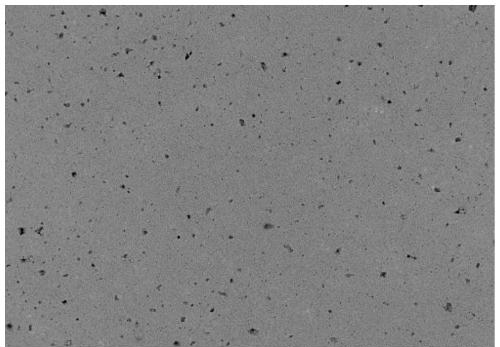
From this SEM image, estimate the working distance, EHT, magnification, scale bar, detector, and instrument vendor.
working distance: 9.4 mm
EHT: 15 kV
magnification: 0.5 K X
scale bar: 100000 nm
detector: BSE M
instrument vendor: JEOL